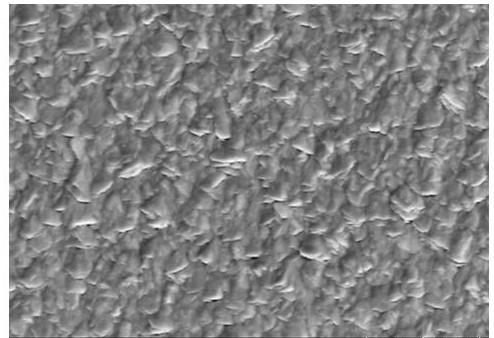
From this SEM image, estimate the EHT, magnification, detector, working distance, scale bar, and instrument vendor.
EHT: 5 kV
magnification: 30 K X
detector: SE(UL)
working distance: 14.8 mm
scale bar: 1000 nm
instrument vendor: Hitachi